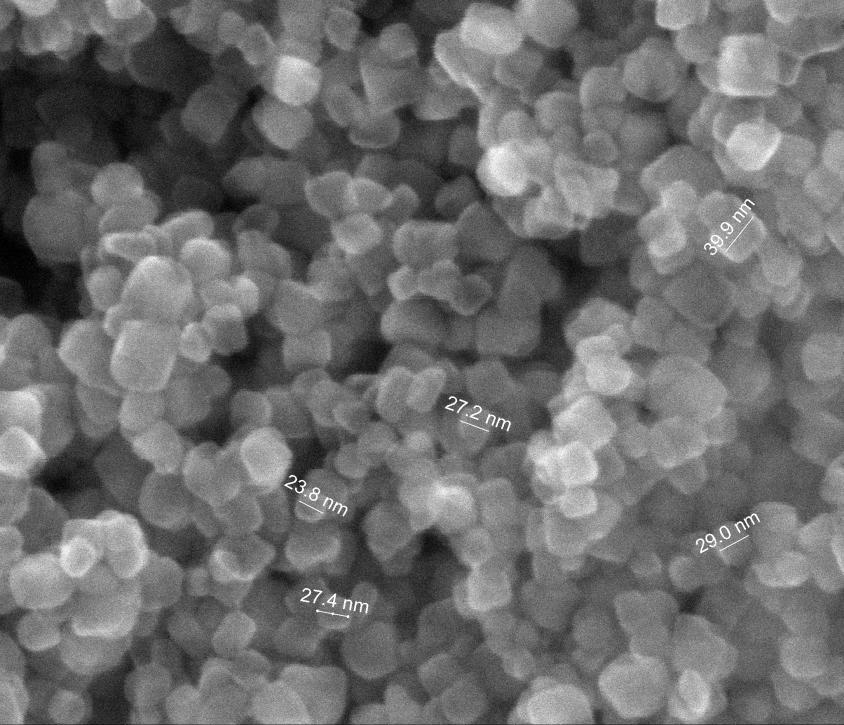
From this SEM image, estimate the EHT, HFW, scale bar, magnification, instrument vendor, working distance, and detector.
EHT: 30 kV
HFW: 0.746 µm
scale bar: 200 nm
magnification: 400 K X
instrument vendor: FEI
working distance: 9.7 mm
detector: ETD